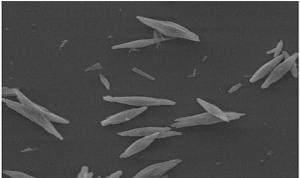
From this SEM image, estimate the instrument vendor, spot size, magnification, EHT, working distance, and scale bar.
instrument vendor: FEI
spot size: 3.8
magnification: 4 K X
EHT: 20 kV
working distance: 9.7 mm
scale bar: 20000 nm